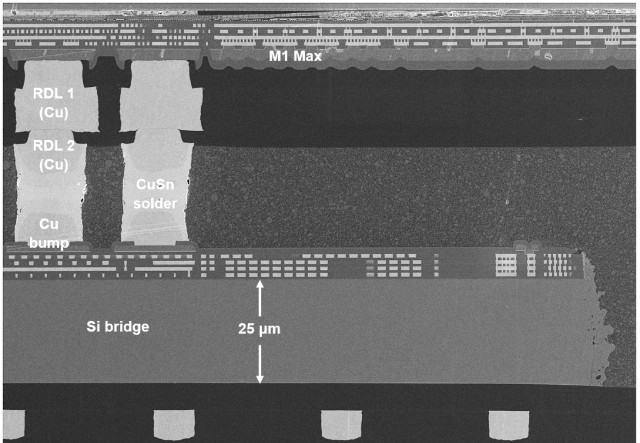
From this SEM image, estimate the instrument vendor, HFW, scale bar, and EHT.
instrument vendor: Zeiss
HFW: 151.1 µm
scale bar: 20000 nm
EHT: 5 kV